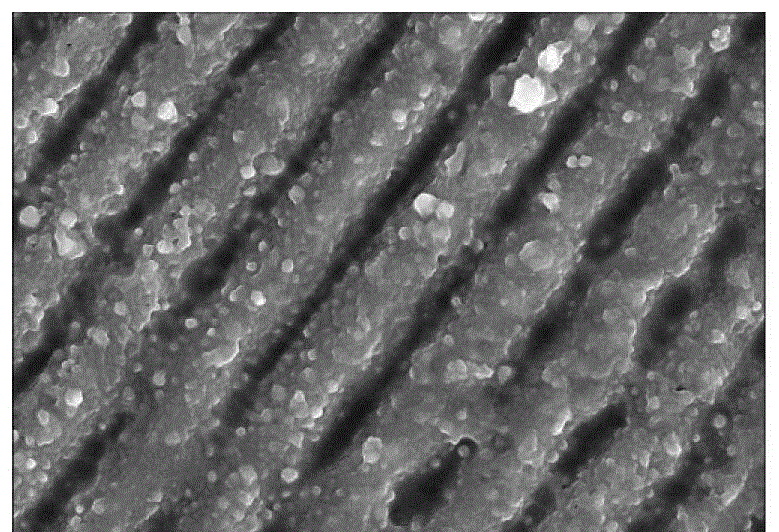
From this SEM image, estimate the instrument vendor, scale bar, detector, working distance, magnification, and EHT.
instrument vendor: Hitachi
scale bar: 2000 nm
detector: SE(UL)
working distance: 8.9 mm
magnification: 50 K X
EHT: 10 kV